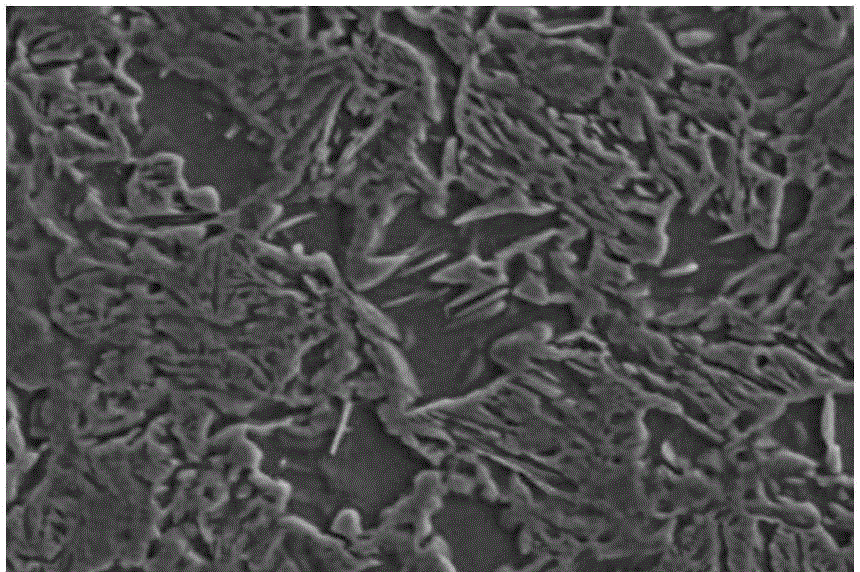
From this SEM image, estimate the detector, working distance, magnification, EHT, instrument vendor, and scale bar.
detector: SE(M)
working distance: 15 mm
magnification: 2 K X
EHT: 20 kV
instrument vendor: Hitachi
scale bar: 20000 nm